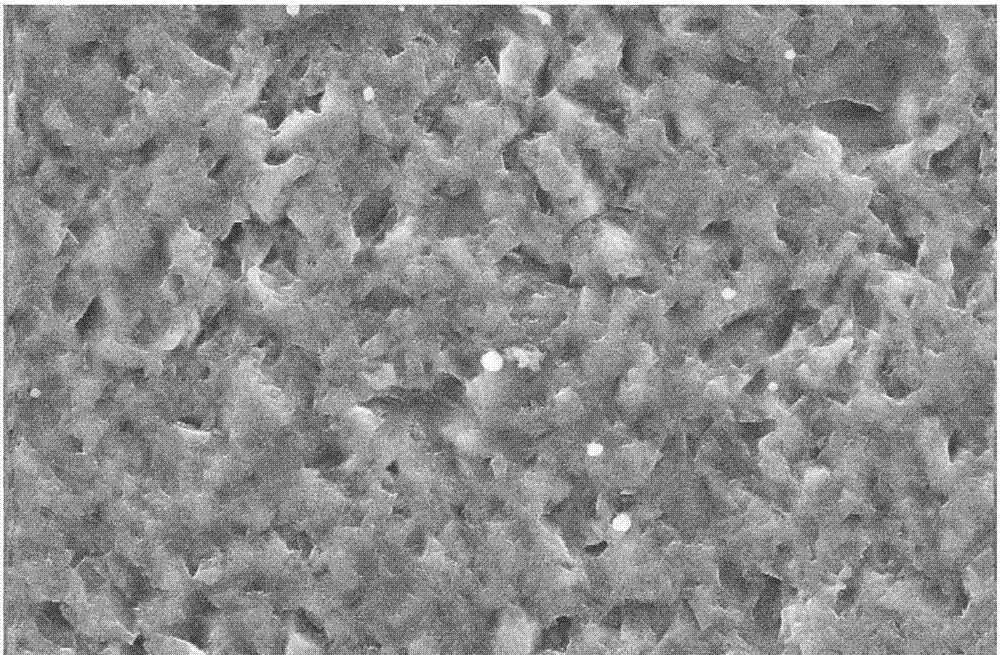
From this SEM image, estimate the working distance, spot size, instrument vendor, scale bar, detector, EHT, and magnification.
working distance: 6.1 mm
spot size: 3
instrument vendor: FEI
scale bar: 20000 nm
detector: SE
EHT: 20 kV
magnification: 1.5 K X